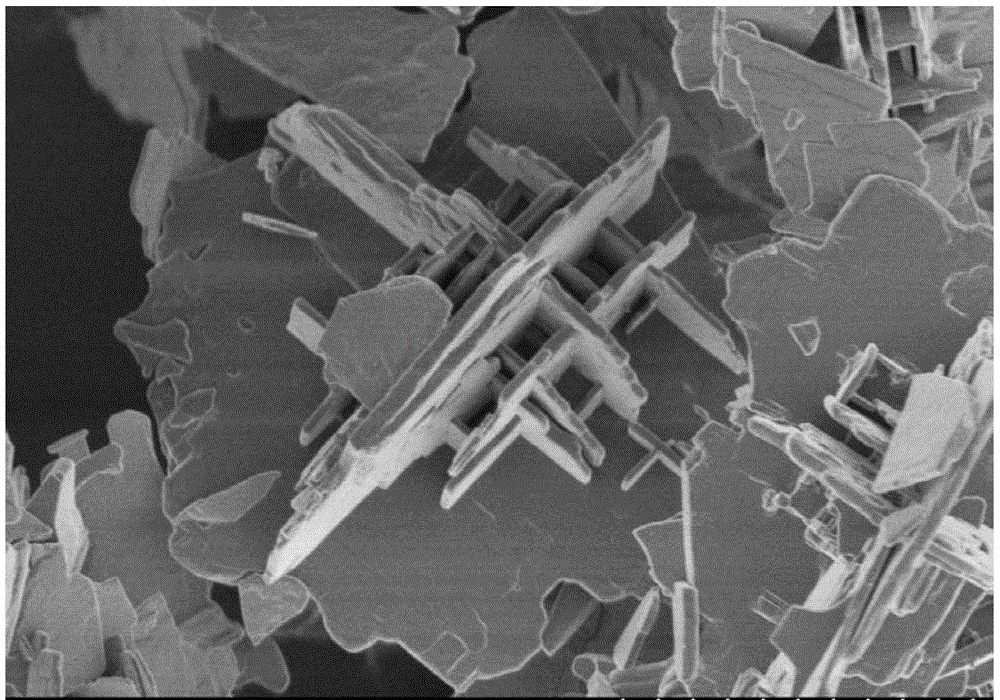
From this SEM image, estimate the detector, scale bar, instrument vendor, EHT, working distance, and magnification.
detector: SE(M)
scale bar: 5000 nm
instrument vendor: Hitachi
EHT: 1 kV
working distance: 8.1 mm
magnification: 9 K X